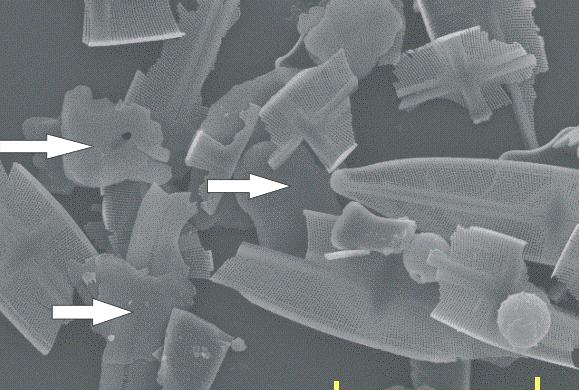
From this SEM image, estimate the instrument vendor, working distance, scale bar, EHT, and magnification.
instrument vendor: JEOL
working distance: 15.1 mm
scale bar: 50000 nm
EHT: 15 kV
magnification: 0.9 K X